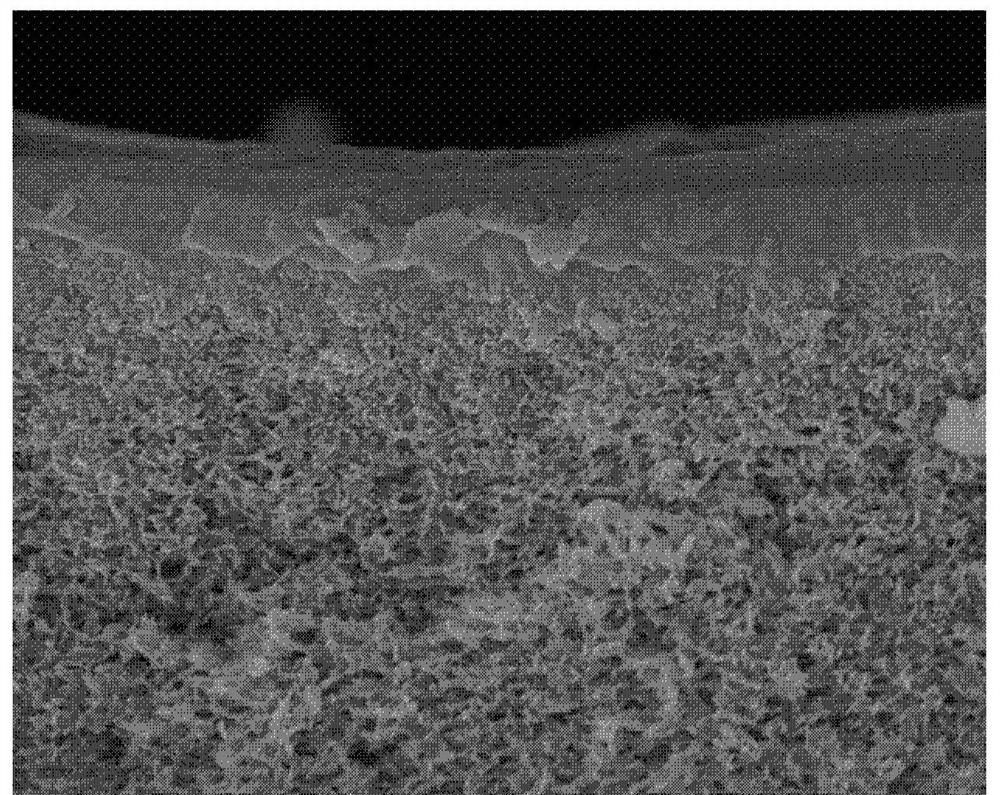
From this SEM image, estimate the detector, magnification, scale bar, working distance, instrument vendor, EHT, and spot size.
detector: TLD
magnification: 30 K X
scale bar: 2000 nm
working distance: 6.4 mm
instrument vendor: FEI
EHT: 15 kV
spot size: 4.5